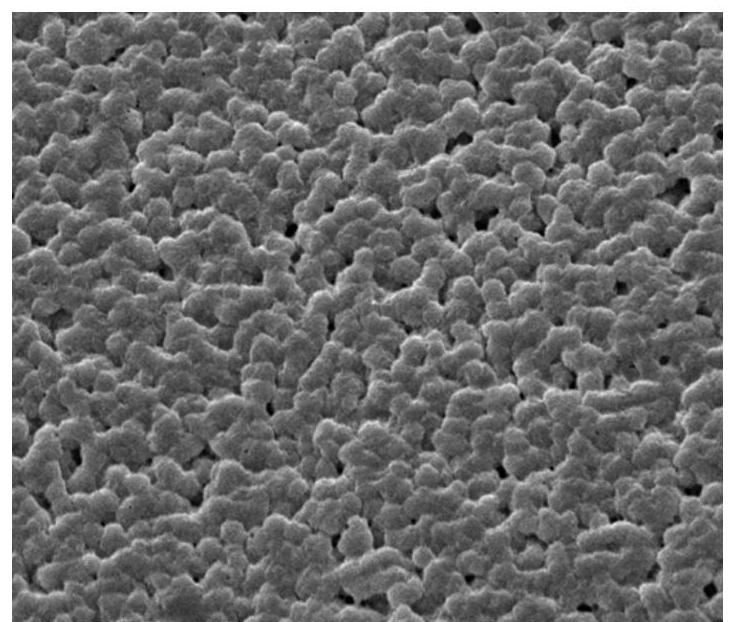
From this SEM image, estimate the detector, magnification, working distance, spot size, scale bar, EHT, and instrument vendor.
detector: ETD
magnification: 2 K X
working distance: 11.4 mm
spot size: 5.5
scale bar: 20000 nm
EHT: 12.5 kV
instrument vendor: FEI